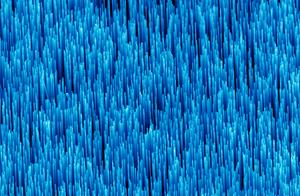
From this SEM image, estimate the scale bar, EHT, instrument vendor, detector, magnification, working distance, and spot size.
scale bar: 2000 nm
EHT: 10 kV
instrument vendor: FEI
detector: SE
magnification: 8.107 K X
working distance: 13 mm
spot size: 3